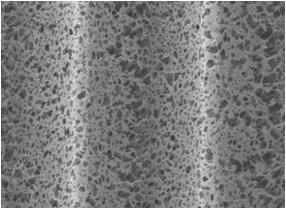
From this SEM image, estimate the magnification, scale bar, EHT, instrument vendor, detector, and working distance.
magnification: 2.5 K X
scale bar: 10000 nm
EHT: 10 kV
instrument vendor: JEOL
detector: LEI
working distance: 8 mm